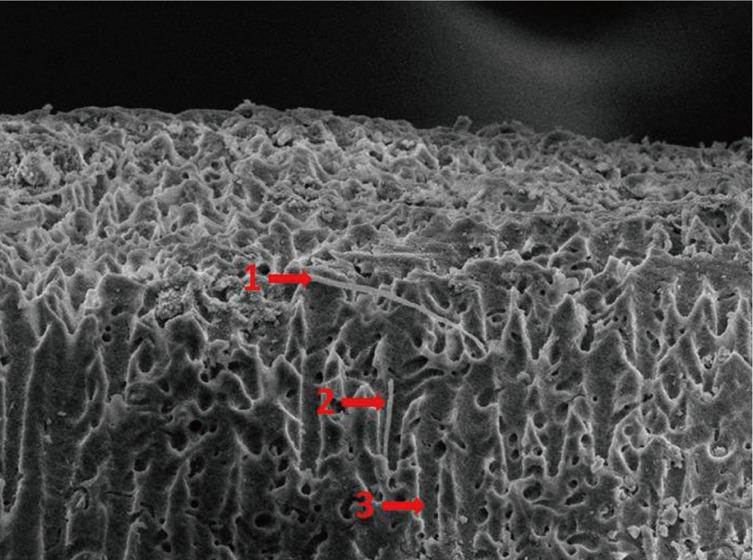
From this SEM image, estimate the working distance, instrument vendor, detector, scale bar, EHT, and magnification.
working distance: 9.7 mm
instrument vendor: JEOL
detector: SEI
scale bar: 10000 nm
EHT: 10 kV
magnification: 1 K X